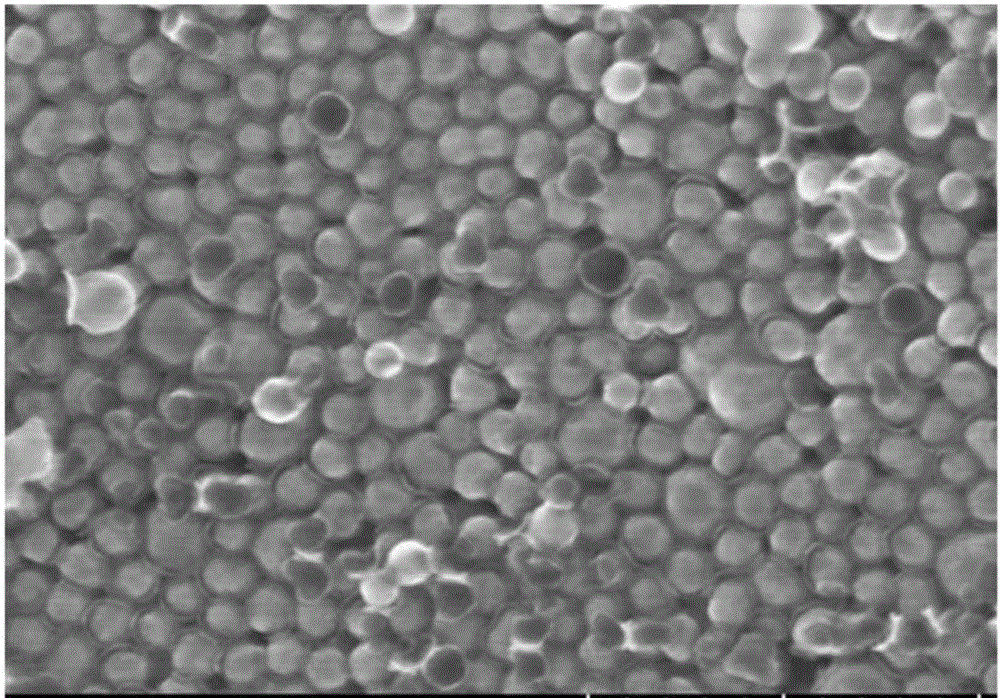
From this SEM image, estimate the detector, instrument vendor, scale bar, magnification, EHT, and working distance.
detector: SE(UL)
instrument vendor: Hitachi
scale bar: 500 nm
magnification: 100 K X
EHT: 15 kV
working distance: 5.4 mm